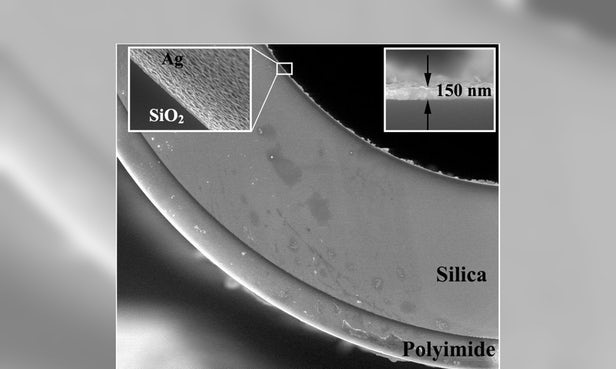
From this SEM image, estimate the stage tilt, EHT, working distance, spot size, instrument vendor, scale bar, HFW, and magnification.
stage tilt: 0°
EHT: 20 kV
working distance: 9.8 mm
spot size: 4.5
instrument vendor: FEI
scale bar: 100000 nm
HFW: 298 µm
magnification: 0.5 K X